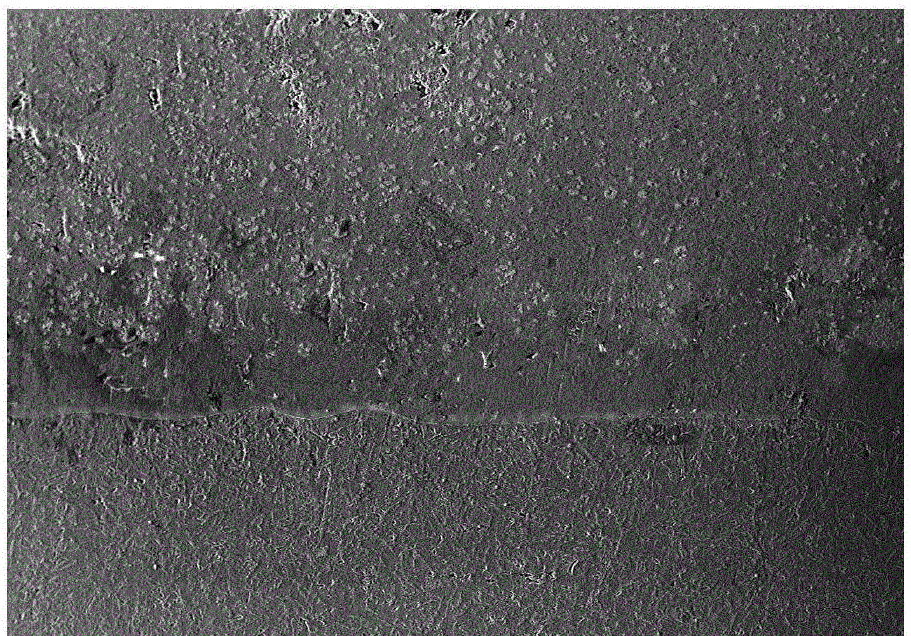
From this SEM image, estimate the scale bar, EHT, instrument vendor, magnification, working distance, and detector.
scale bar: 50000 nm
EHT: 20 kV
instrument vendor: Hitachi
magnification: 0.1 K X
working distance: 15.3 mm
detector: SE(M)